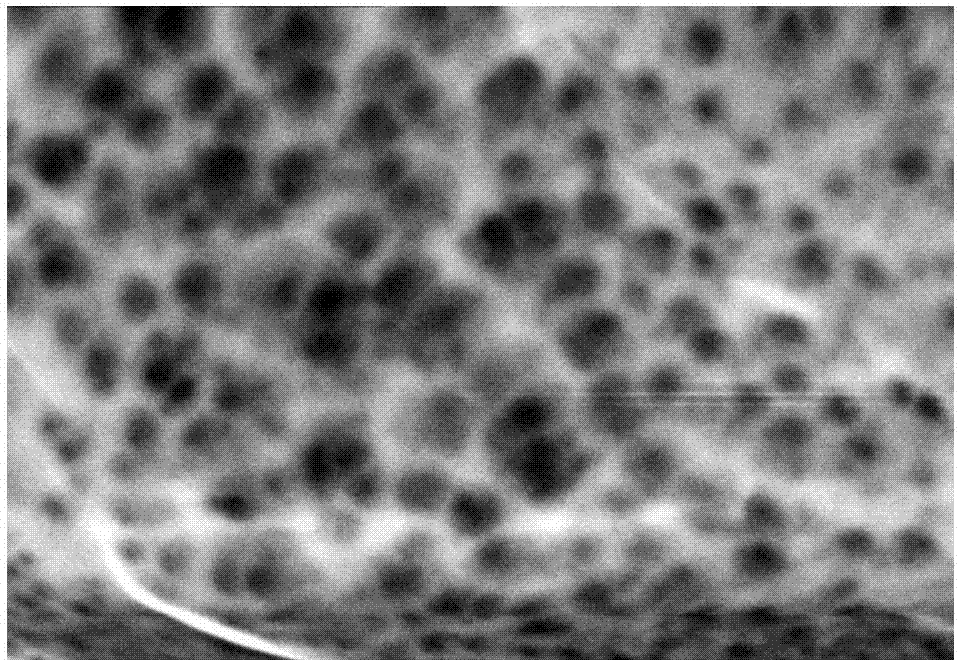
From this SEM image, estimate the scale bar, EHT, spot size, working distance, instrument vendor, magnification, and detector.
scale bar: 1000 nm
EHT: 15 kV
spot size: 16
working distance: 6.3 mm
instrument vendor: other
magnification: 10 K X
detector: SEI